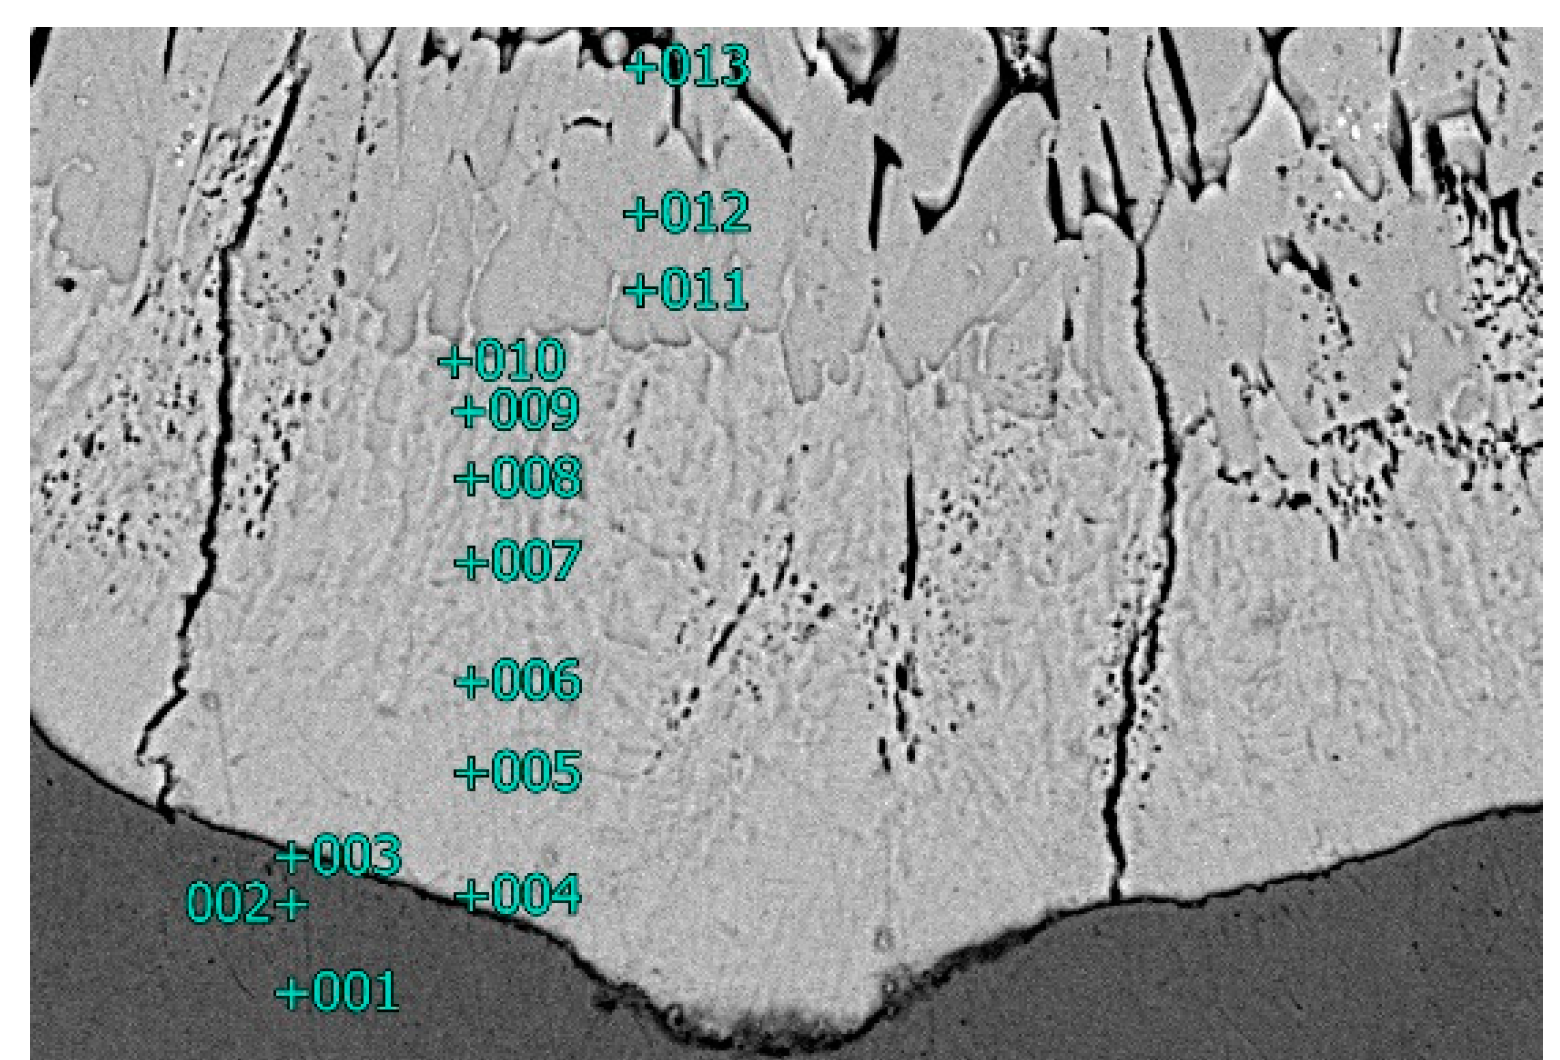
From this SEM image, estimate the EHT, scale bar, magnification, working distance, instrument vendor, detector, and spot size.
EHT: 15 kV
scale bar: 10000 nm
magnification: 2 K X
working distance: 10 mm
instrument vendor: JEOL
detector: BEC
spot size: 40